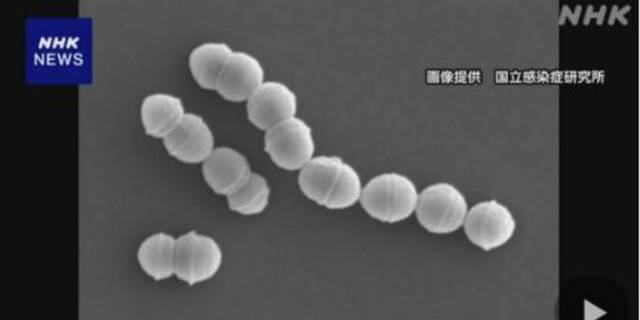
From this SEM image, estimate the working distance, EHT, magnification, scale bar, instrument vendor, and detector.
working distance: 12.1 mm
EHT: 5 kV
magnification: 20 K X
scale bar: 2000 nm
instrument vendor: Hitachi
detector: SE